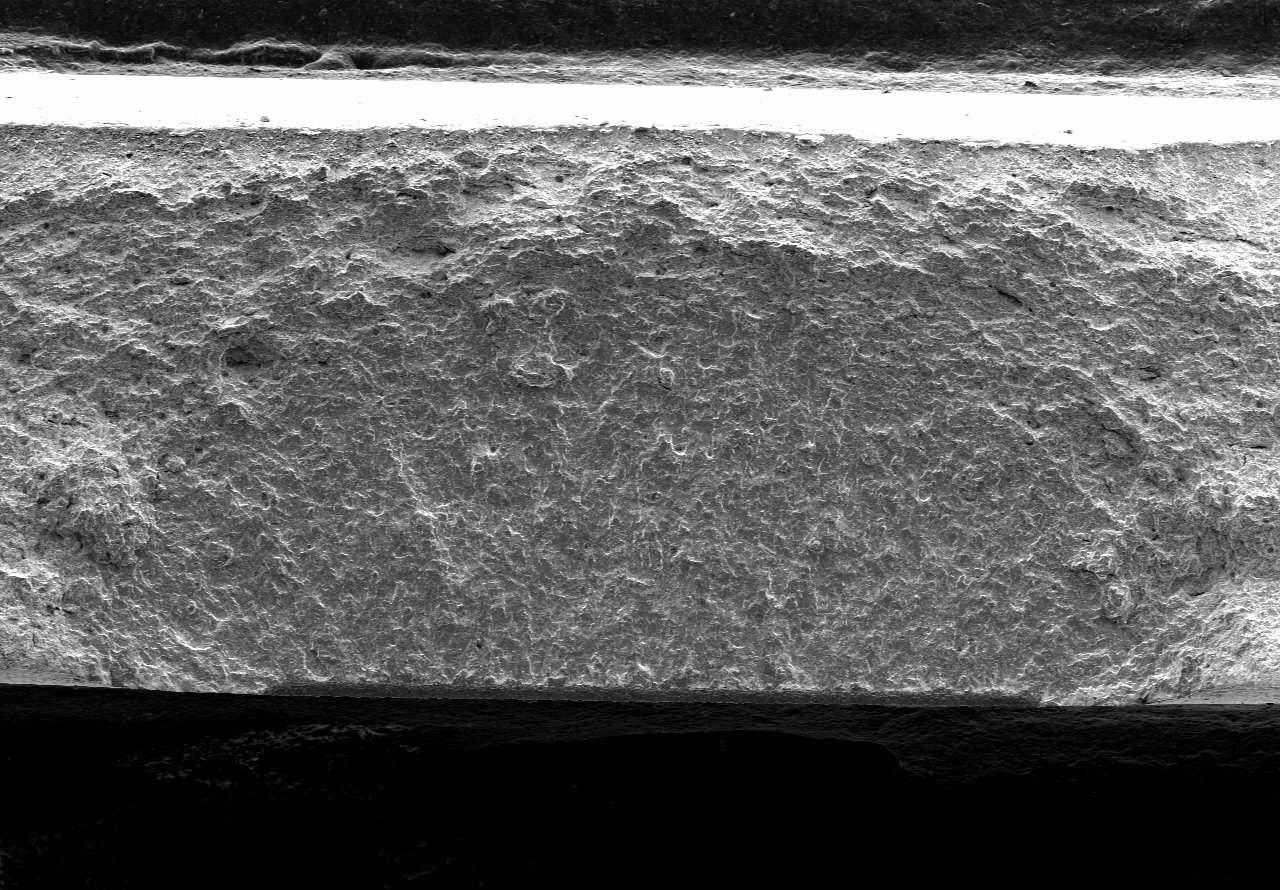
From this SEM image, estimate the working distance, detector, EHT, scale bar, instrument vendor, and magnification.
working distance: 17.8 mm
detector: SE(M)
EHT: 8 kV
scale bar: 1000000 nm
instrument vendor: Hitachi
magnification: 0.04 K X